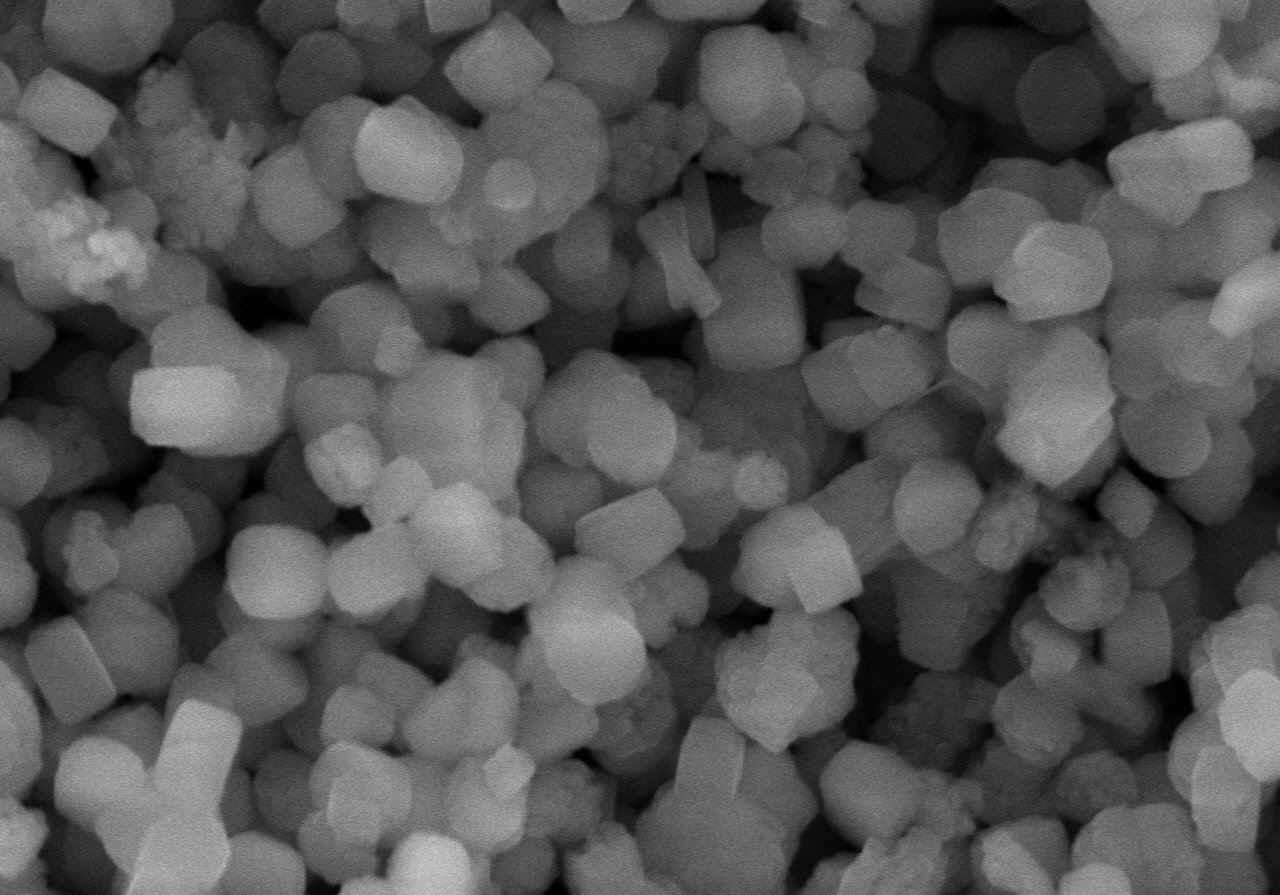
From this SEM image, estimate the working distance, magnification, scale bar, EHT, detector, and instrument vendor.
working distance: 8 mm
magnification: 20 K X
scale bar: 2000 nm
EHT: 10 kV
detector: SE(M)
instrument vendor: Hitachi